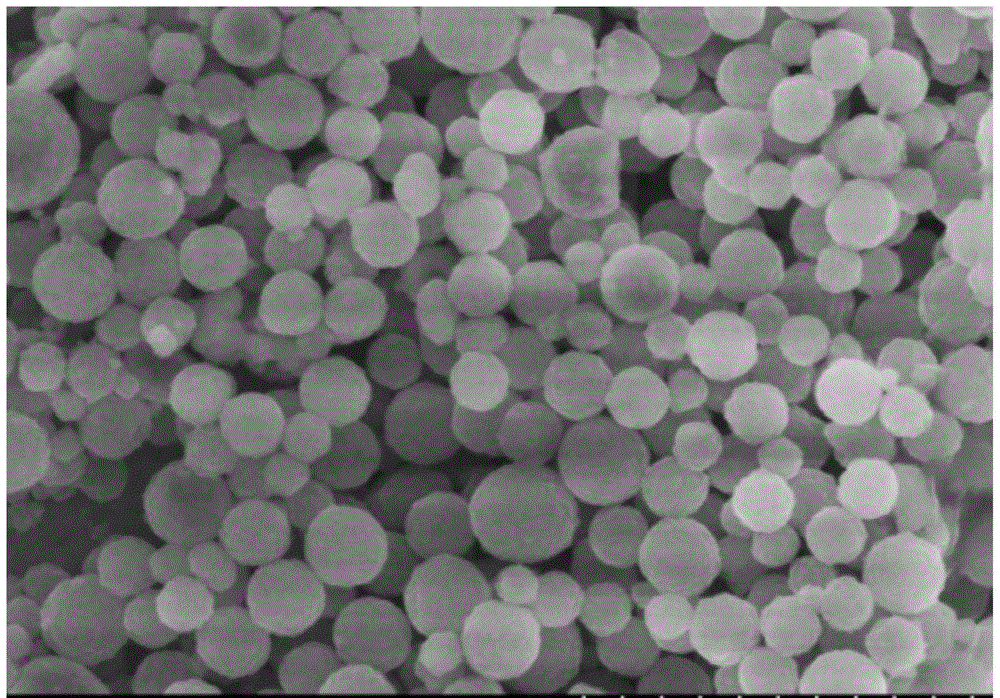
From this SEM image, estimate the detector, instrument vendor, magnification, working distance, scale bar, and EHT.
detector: SE(M,LA0)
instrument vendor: Hitachi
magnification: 25 K X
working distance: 10.7 mm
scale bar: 2000 nm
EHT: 5 kV